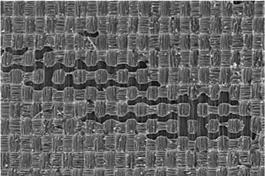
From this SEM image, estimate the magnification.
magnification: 0.03 K X